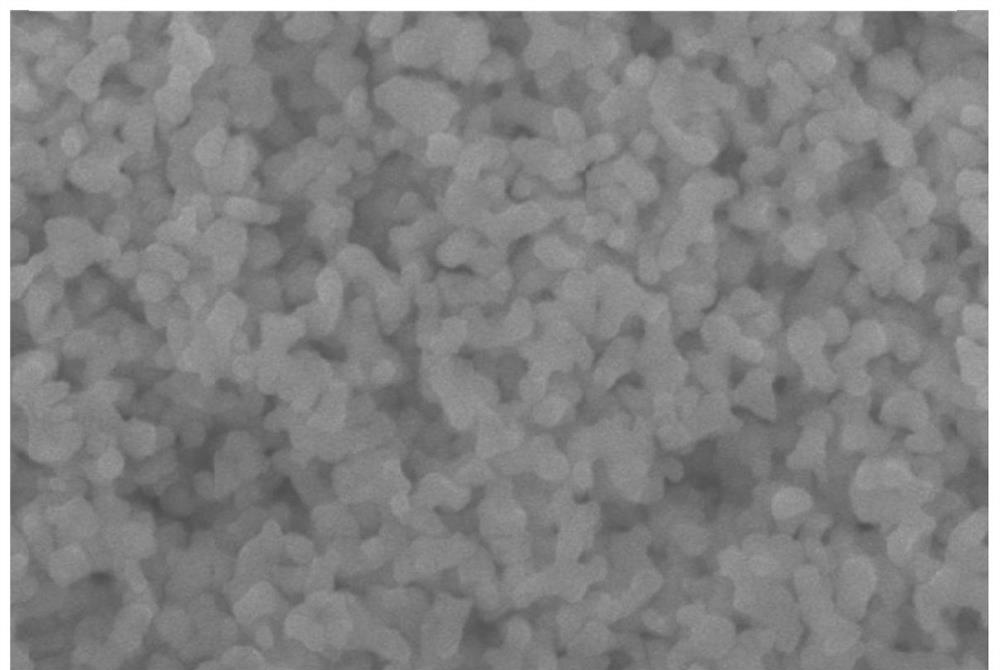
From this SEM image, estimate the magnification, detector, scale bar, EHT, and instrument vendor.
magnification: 20 K X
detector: SEI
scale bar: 1000 nm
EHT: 20 kV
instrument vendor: JEOL